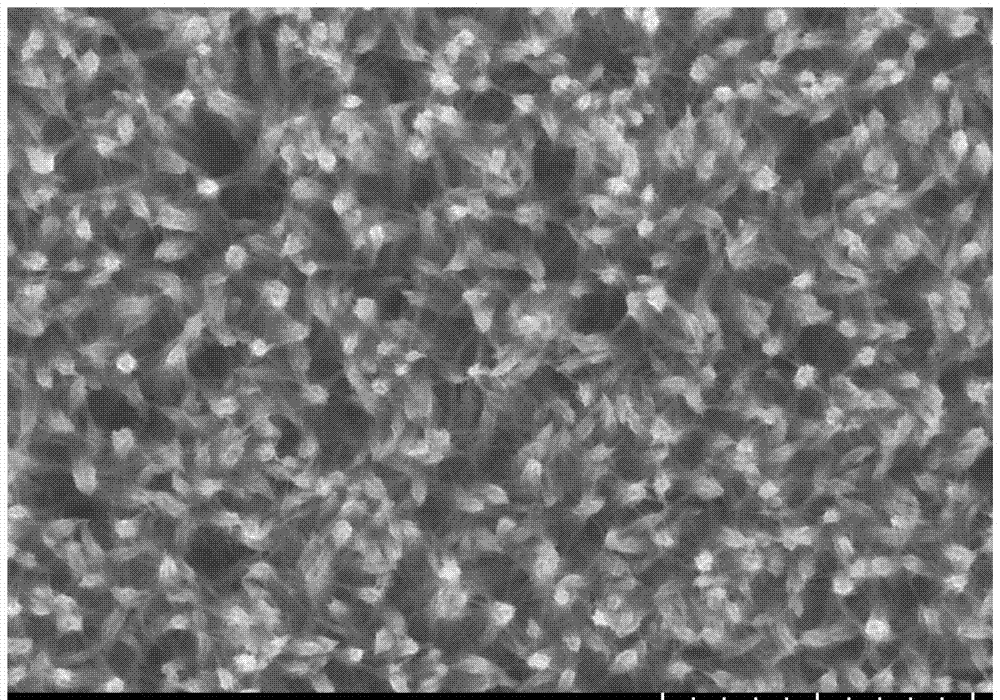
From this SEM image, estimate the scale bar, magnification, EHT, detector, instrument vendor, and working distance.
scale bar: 2000 nm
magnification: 20 K X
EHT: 15 kV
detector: SE(UL)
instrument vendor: Hitachi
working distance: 8.9 mm